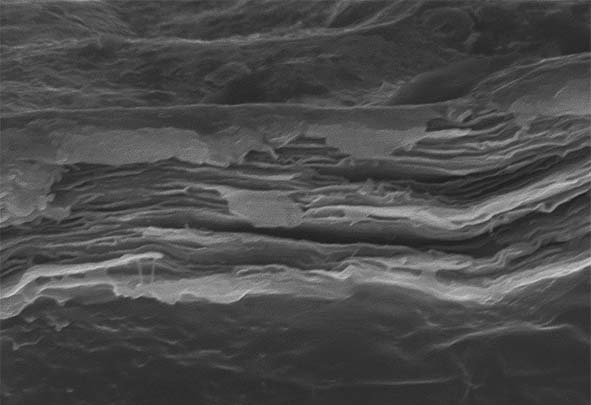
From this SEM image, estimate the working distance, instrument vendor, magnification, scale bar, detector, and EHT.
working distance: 15.2 mm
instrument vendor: Hitachi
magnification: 13 K X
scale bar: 4000 nm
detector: SE(M)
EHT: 15 kV